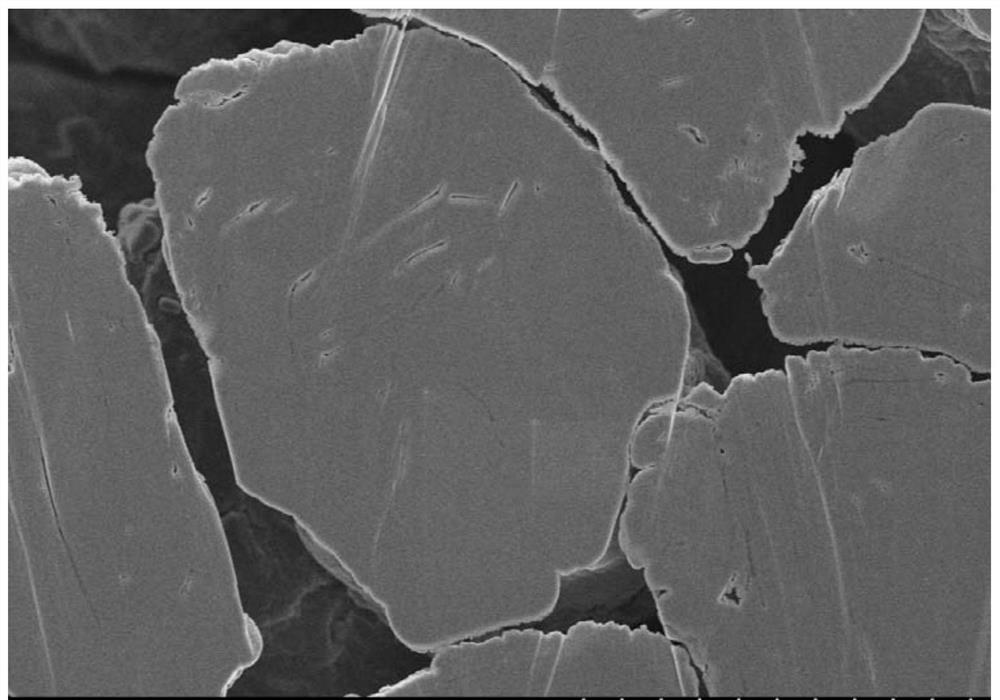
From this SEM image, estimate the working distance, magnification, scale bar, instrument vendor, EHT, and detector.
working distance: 5 mm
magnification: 5 K X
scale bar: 10000 nm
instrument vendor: Hitachi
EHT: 3 kV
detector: SE(M)